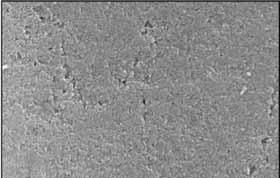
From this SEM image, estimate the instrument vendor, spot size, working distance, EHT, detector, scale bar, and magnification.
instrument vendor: FEI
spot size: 3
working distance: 10.1 mm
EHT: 25 kV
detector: SE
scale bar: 1000 nm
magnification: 20 K X